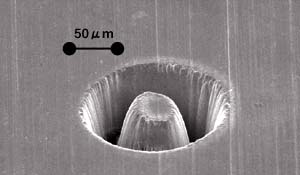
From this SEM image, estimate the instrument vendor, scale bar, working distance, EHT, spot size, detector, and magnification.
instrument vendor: FEI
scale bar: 50000 nm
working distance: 11.6 mm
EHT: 25 kV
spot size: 3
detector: SE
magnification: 0.4 K X